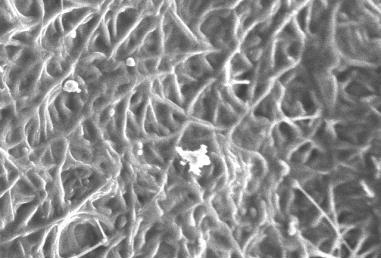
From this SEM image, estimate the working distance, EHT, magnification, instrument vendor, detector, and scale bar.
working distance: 8 mm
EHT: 10 kV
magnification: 1.5 K X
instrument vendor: JEOL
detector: SEI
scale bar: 10000 nm